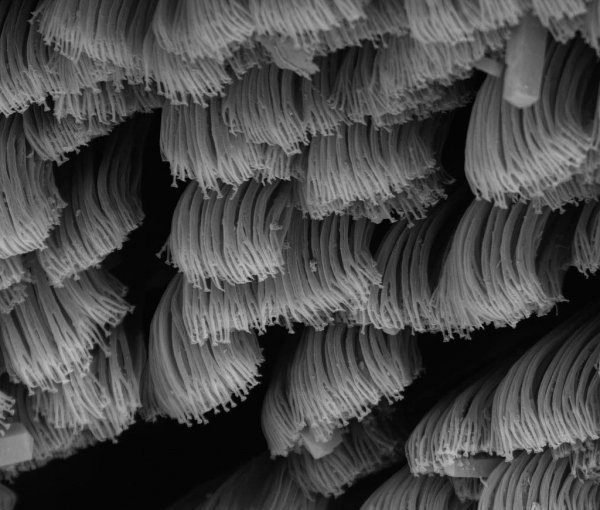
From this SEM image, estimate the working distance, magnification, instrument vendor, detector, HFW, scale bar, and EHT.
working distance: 8.2 mm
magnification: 10 K X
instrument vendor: FEI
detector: BSED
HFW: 29.8 µm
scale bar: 5000 nm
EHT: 15 kV